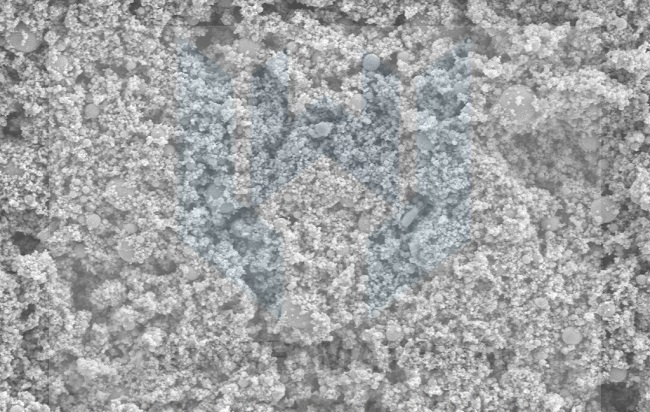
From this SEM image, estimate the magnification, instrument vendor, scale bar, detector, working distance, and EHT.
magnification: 10 K X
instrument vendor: Zeiss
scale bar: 1000 nm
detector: SE2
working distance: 8.6 mm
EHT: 5 kV